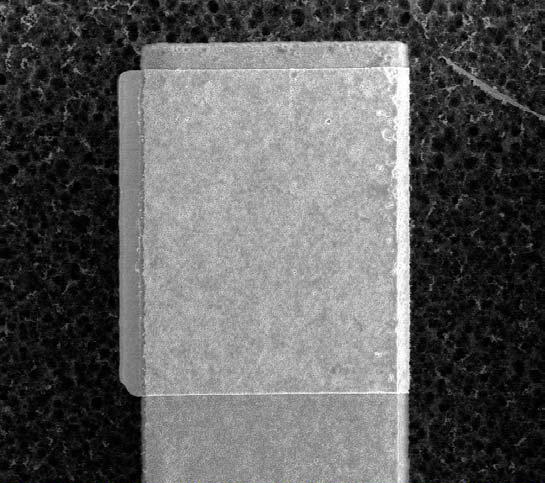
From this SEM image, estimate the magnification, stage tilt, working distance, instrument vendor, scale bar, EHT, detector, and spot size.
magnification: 3.5 K X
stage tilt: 45°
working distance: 8.83 mm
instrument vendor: FEI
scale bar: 10000 nm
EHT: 15 kV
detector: CDM-E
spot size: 3